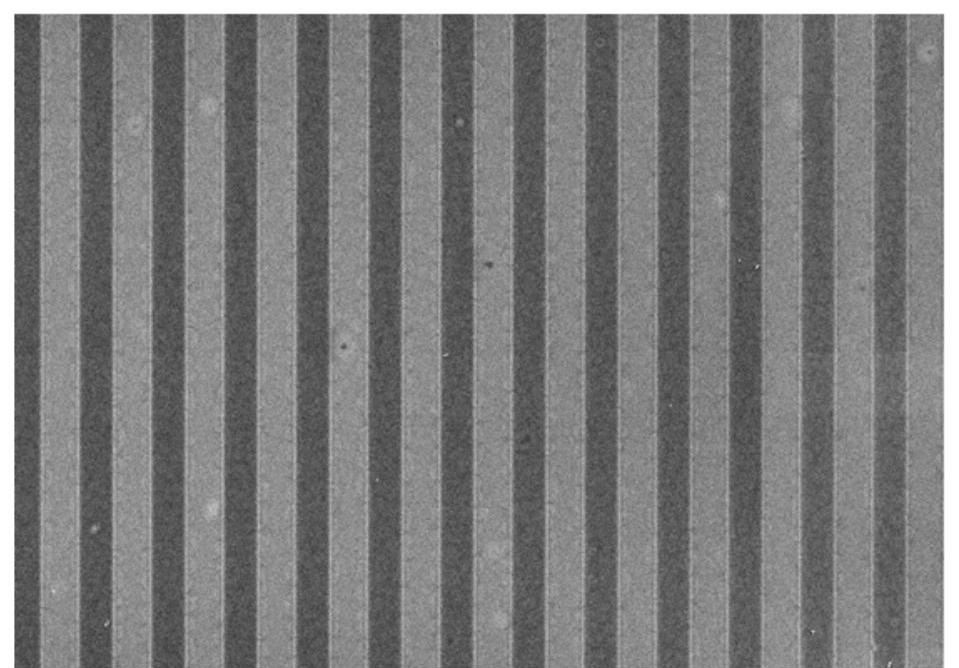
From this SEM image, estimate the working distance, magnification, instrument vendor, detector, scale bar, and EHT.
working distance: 10.5 mm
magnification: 0.5 K X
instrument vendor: Hitachi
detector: SE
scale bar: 100000 nm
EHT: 5 kV